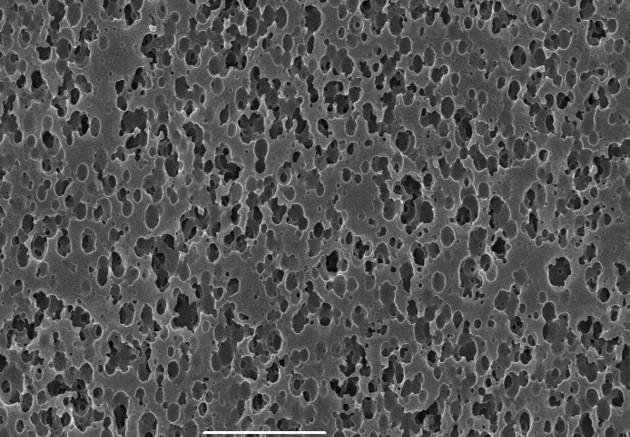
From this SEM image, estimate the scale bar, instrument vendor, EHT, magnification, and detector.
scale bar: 5000 nm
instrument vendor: JEOL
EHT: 15 kV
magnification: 5 K X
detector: SEI